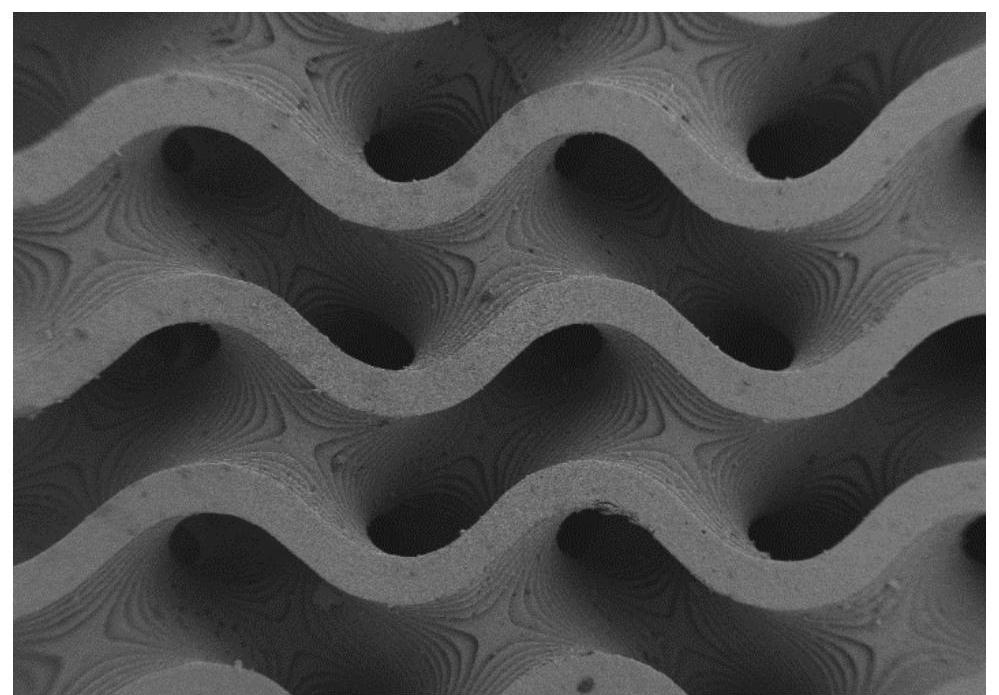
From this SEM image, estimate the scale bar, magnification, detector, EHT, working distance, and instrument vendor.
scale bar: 200000 nm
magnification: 0.031 K X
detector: SE2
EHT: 3 kV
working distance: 6.5 mm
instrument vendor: Zeiss